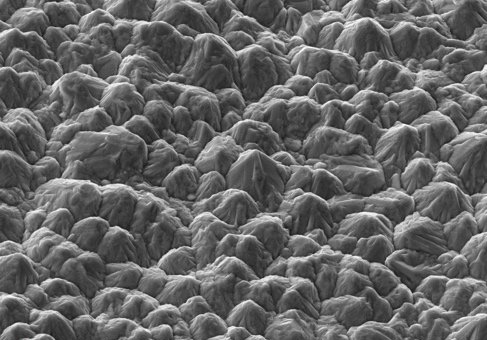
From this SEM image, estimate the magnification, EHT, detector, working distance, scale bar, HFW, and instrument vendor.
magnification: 3 K X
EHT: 15 kV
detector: ETD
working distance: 10.2 mm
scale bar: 10000 nm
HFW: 42.3 µm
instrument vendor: Thermo